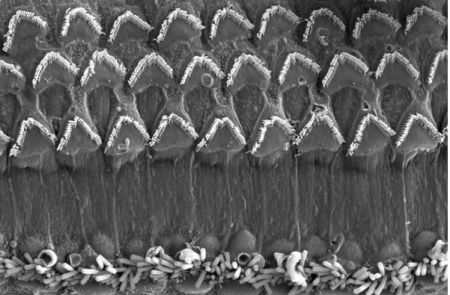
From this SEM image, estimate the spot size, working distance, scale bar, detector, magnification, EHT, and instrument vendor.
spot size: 3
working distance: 7.4 mm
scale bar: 20000 nm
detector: SE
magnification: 2.5 K X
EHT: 10 kV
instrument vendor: FEI